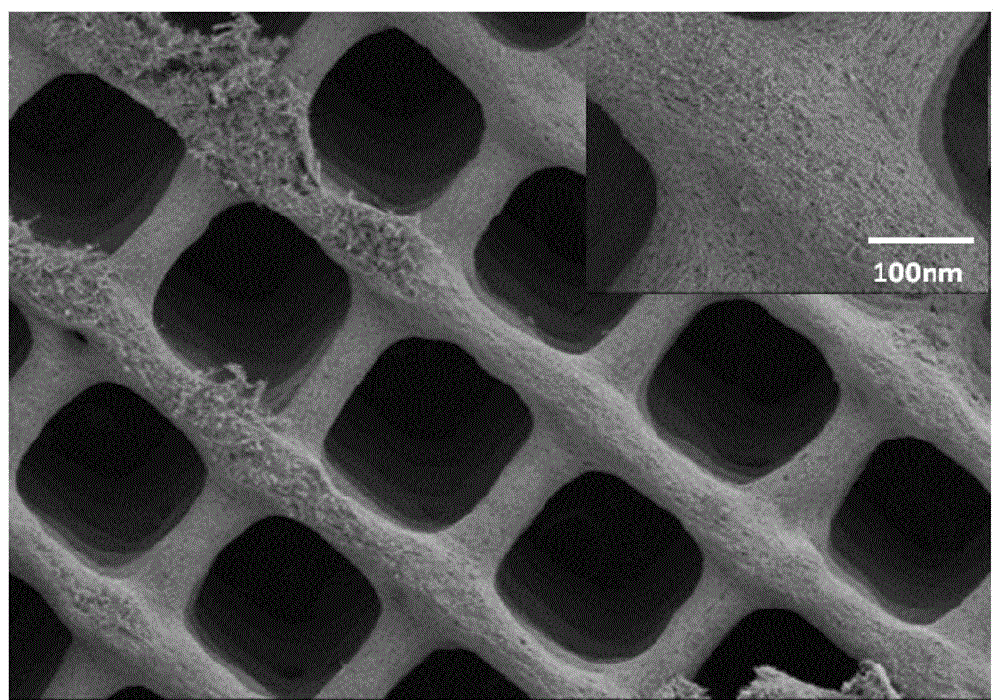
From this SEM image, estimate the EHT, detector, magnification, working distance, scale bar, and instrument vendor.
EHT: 5 kV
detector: SE(M)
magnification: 0.05 K X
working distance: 8.6 mm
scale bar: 1000000 nm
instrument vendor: Hitachi